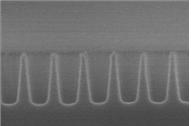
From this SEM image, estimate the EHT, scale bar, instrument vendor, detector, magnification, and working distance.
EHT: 15 kV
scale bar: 200 nm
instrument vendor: Hitachi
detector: SE(U)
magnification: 200 K X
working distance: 6.9 mm